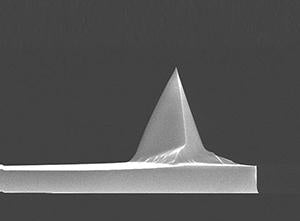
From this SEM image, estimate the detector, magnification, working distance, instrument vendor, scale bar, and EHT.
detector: TLD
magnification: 3.5 K X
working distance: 4.9 mm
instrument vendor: FEI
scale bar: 10000 nm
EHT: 15 kV